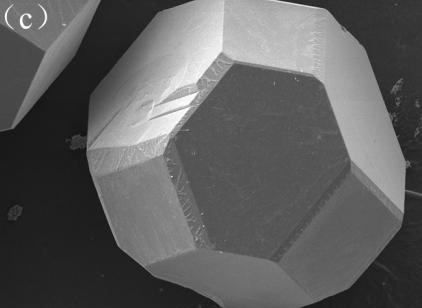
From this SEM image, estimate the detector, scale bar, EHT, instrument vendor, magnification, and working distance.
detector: SEI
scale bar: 100000 nm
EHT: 15 kV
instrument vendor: JEOL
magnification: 0.2 K X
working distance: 8 mm